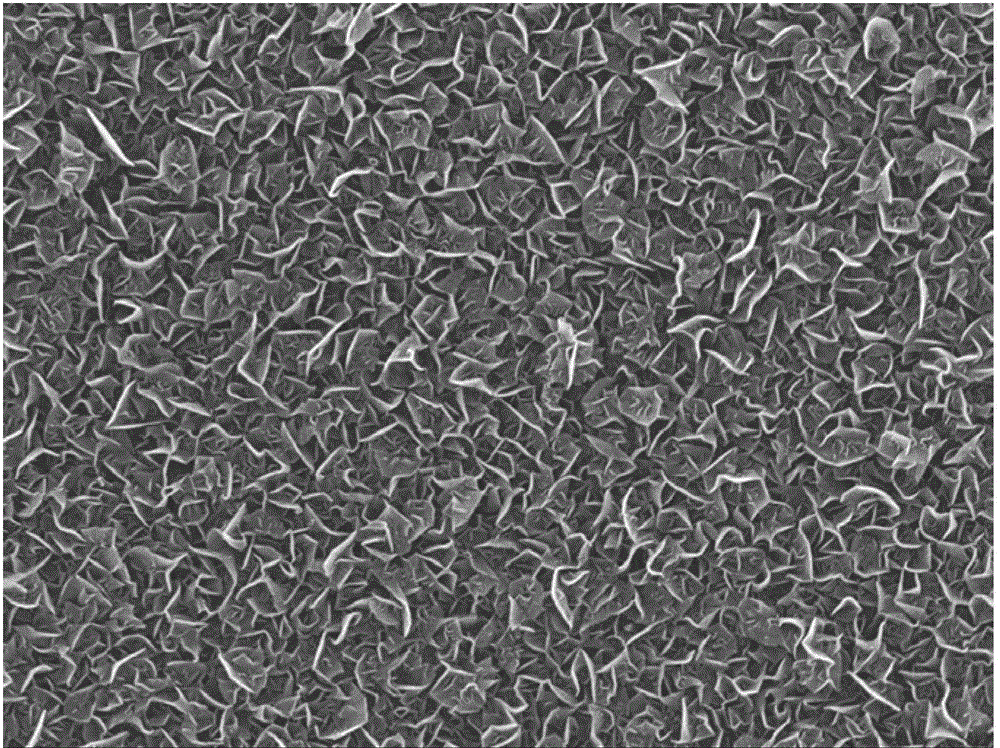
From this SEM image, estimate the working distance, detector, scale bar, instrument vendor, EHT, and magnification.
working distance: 4.7 mm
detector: SEI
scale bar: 1000 nm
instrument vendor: JEOL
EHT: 8 kV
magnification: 20 K X